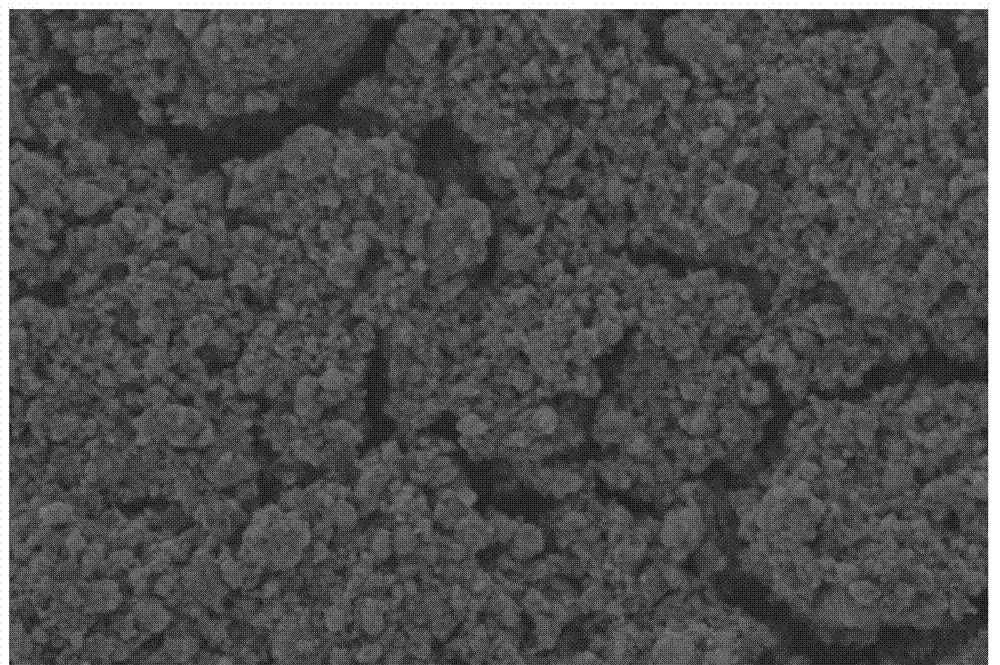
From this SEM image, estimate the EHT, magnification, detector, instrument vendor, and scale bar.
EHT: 10 kV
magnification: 0.7 K X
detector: SEI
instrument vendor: JEOL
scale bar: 20000 nm